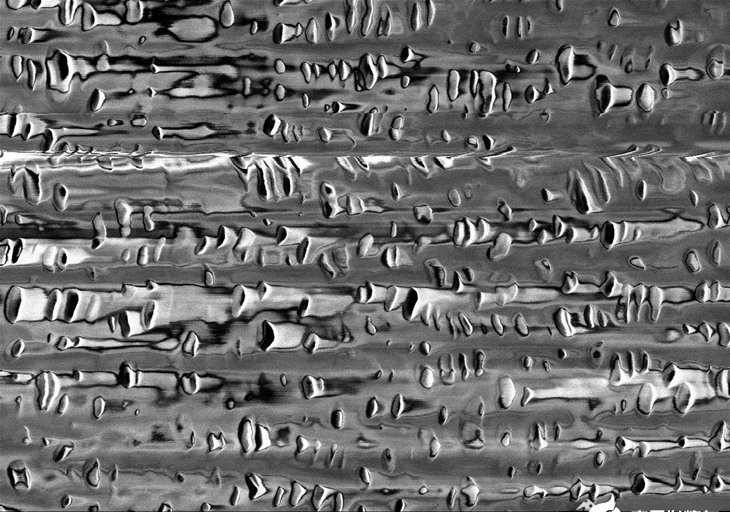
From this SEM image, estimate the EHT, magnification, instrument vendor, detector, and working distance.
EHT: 1 kV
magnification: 35 K X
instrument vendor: Hitachi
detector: SE(U,LA0)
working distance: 1.5 mm